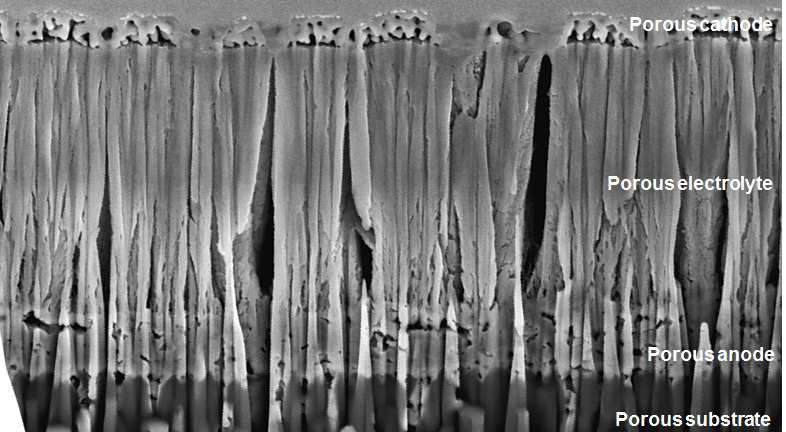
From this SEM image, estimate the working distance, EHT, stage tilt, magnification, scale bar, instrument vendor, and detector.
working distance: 10.1 mm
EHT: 5 kV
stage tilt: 52°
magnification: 50 K X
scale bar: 2000 nm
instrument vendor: FEI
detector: ETD